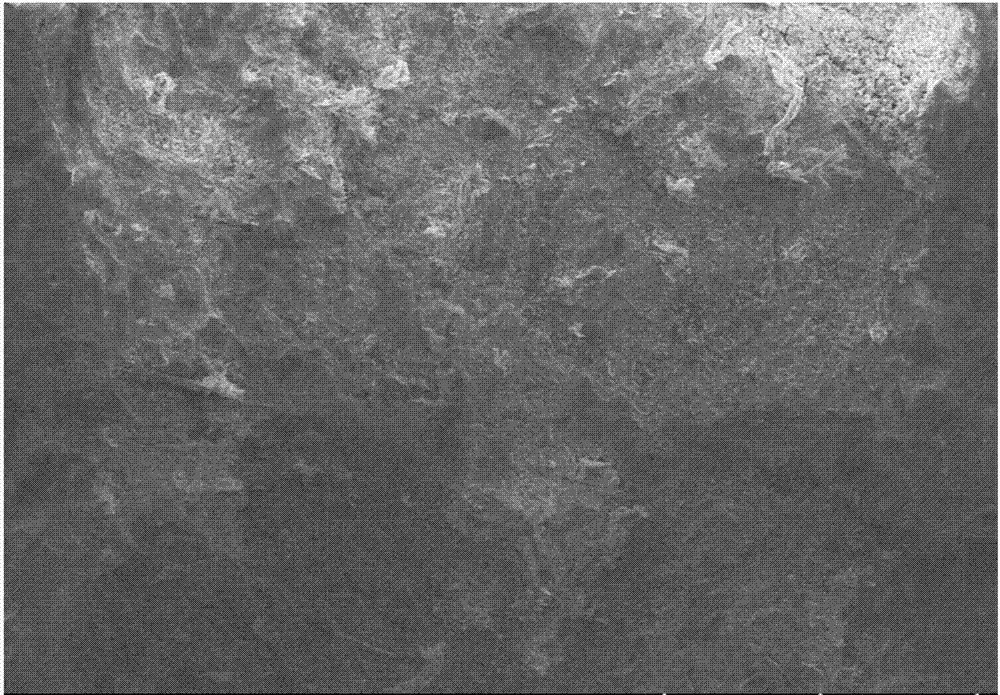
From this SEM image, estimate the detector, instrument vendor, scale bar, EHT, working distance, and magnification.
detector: LM(L)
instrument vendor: Hitachi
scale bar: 500000 nm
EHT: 15 kV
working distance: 14.8 mm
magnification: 0.08 K X